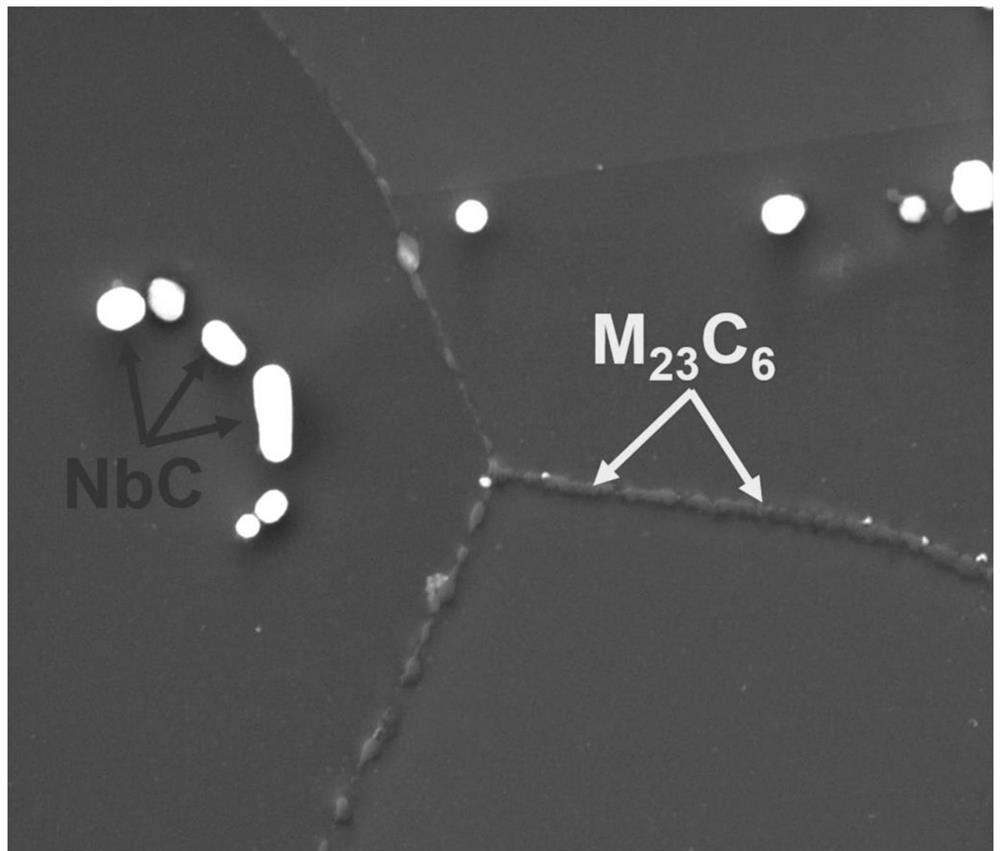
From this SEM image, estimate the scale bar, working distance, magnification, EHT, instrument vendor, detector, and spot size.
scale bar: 10000 nm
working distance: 10.4 mm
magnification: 5 K X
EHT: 20 kV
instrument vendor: FEI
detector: ETD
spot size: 4